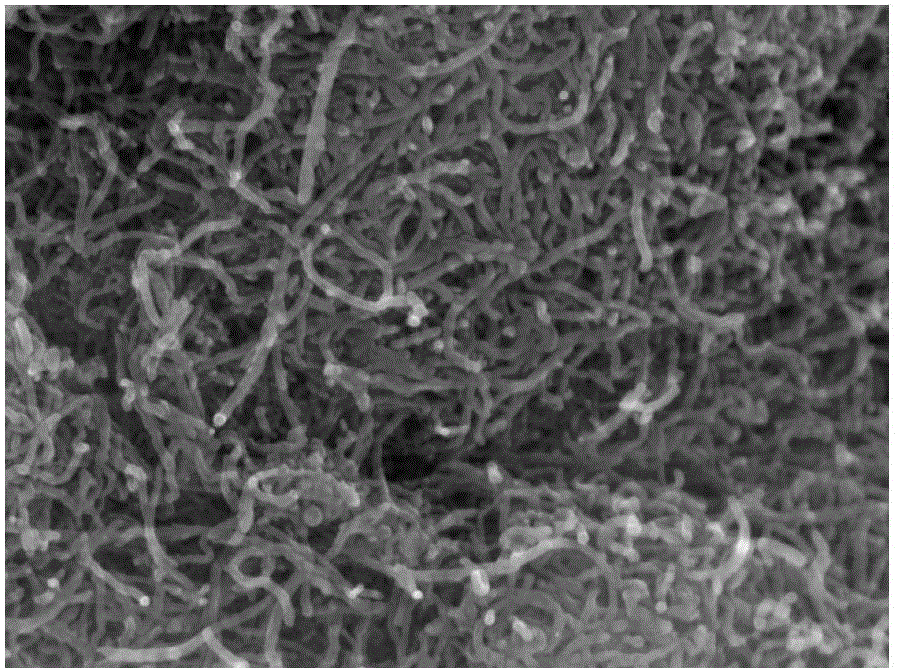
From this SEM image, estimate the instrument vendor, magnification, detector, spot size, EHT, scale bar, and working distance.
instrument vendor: FEI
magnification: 40 K X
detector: TLD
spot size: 5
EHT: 20 kV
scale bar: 500 nm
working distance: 4.9 mm